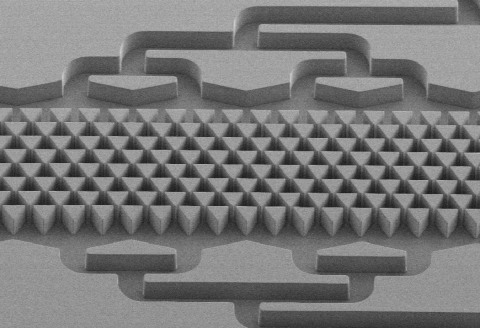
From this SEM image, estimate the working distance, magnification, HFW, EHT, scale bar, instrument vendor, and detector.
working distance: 12.6 mm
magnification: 0.156 K X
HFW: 1956 µm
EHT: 6 kV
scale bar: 100000 nm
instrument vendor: Zeiss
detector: SE2